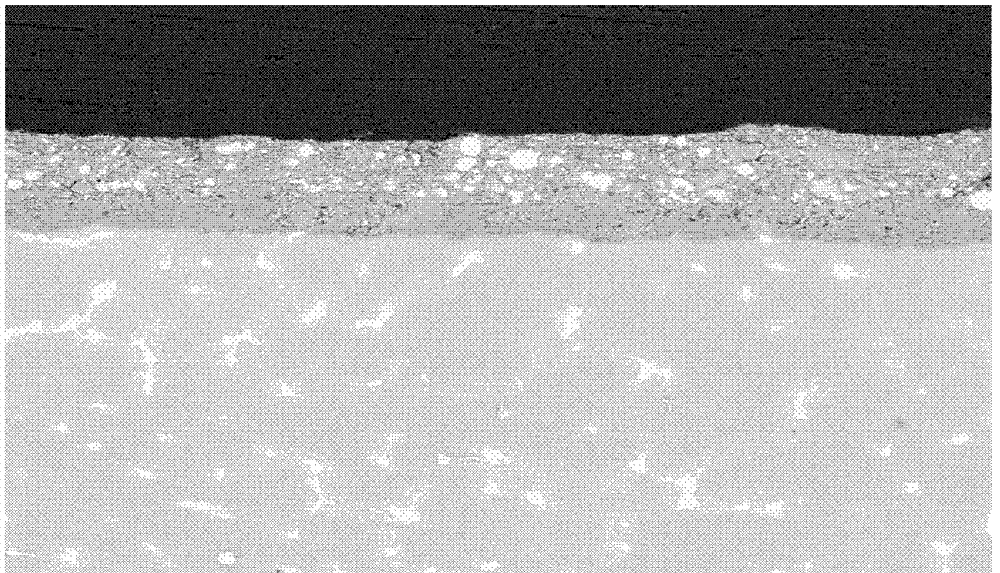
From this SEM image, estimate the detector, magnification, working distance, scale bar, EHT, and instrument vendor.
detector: DualBSD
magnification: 0.5 K X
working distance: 21.4 mm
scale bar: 200000 nm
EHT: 20 kV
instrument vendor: FEI